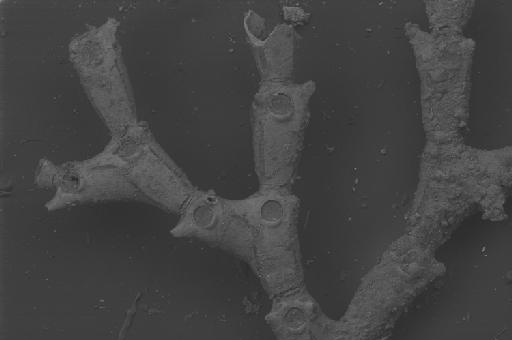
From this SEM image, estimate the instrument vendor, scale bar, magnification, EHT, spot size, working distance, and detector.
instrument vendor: Zeiss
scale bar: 200000 nm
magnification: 0.12 K X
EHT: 20 kV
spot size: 550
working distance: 16 mm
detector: BSD